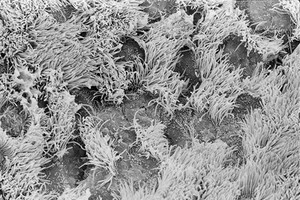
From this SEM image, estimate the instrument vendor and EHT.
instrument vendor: JEOL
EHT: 10 kV